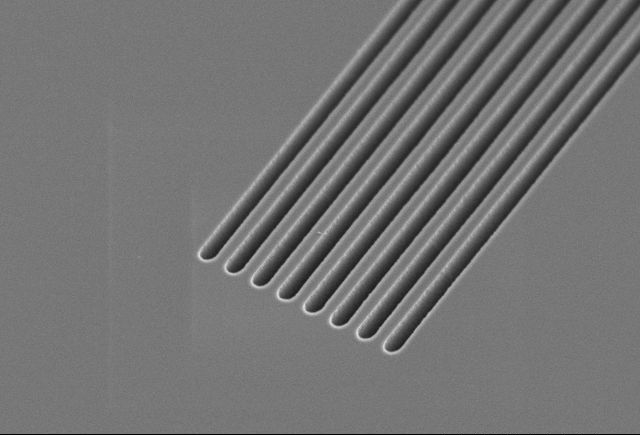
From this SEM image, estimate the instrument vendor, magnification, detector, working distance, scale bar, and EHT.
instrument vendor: JEOL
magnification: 3 K X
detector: SE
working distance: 10.3 mm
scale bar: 10000 nm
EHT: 5 kV